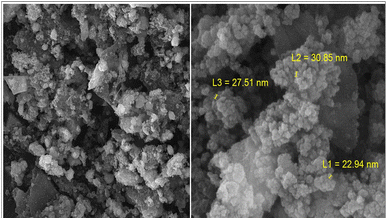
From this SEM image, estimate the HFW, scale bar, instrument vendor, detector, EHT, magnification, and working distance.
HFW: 20.8 µm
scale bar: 5000 nm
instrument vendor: Tescan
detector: SE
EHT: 15 kV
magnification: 10 K X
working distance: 9.89 mm